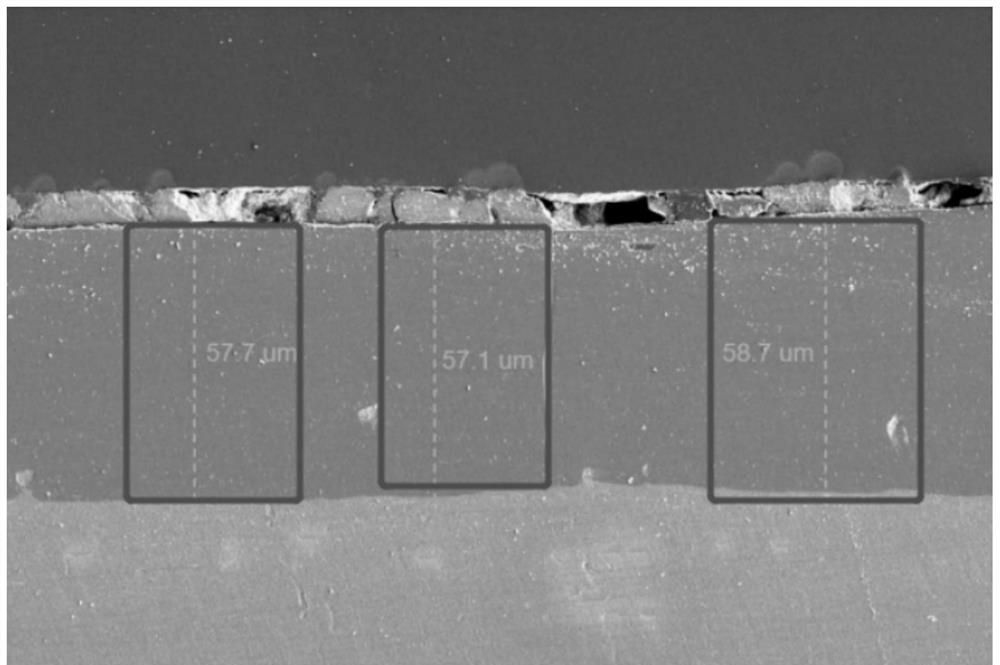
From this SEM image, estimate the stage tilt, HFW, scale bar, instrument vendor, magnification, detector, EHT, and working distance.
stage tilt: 0°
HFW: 207 µm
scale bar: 50000 nm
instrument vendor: FEI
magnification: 1 K X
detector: ETD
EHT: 3 kV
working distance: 7 mm